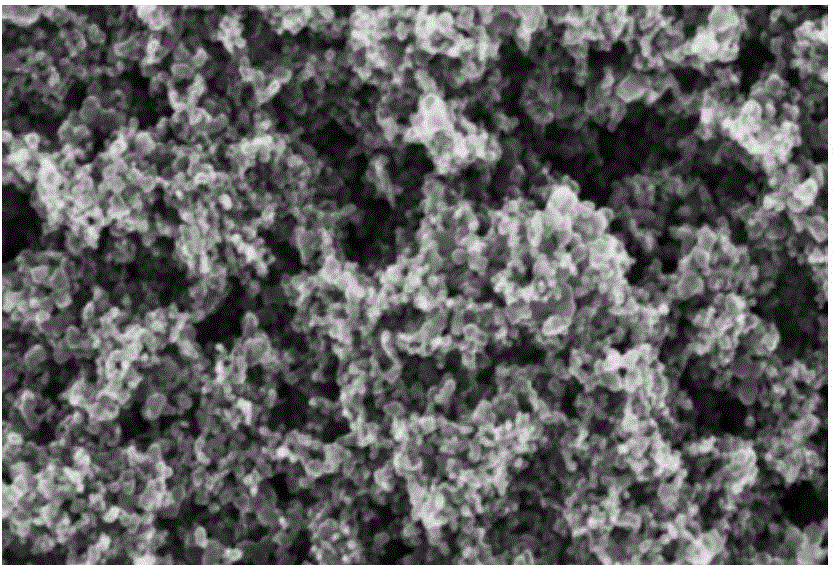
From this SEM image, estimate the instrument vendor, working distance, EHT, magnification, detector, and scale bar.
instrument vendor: Zeiss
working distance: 5.3 mm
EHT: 20 kV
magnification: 50 K X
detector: InLens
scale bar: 100 nm